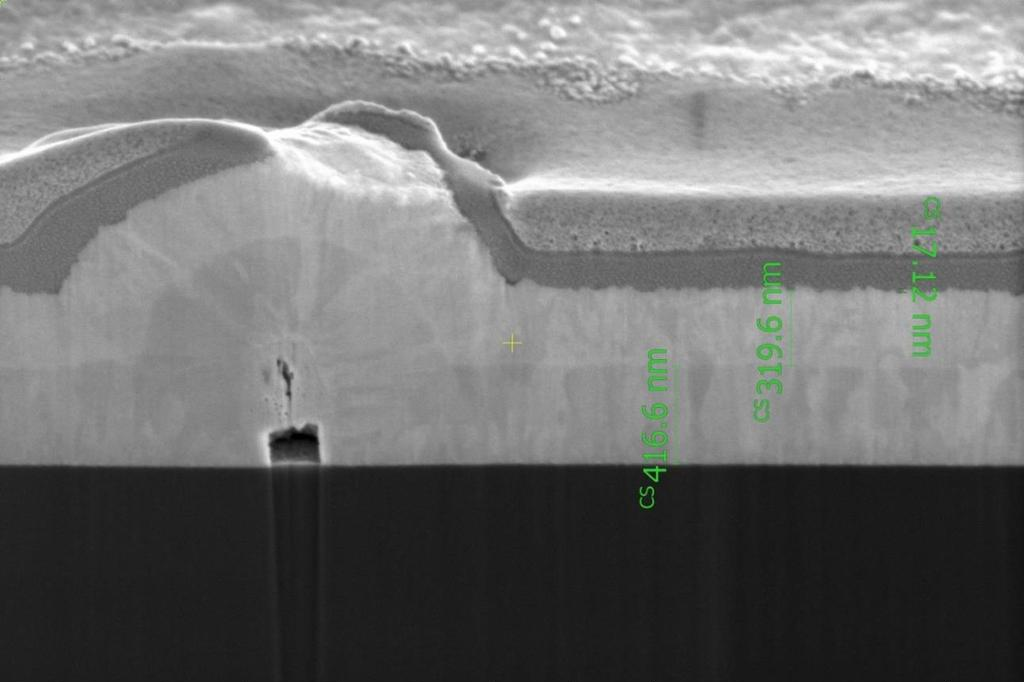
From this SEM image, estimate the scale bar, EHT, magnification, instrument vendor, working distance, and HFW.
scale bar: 1000 nm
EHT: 2 kV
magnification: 120 K X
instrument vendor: FEI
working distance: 3.9 mm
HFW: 3.45 µm